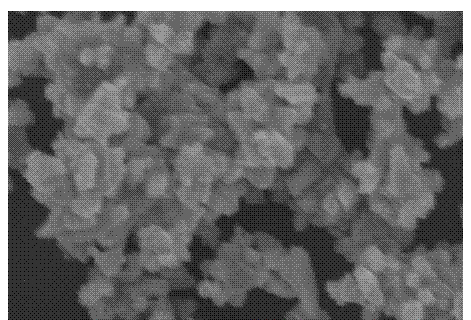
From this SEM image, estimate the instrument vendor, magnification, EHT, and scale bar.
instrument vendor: other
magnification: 3 K X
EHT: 25 kV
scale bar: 10000 nm